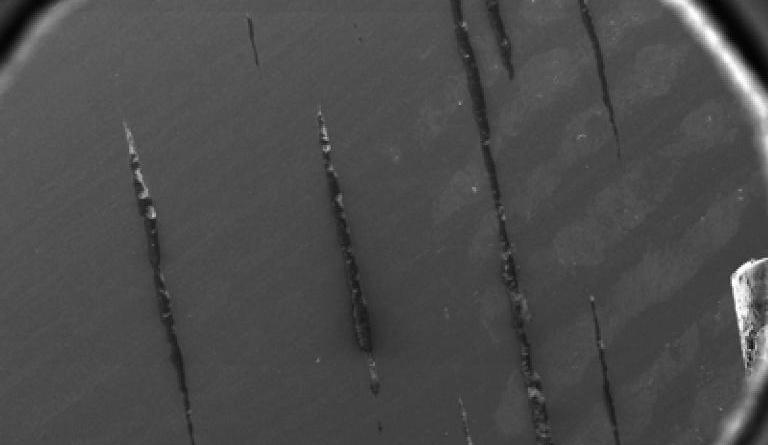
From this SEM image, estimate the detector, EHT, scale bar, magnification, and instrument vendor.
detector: SEI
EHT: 30 kV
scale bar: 500000 nm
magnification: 0.03 K X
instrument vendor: JEOL